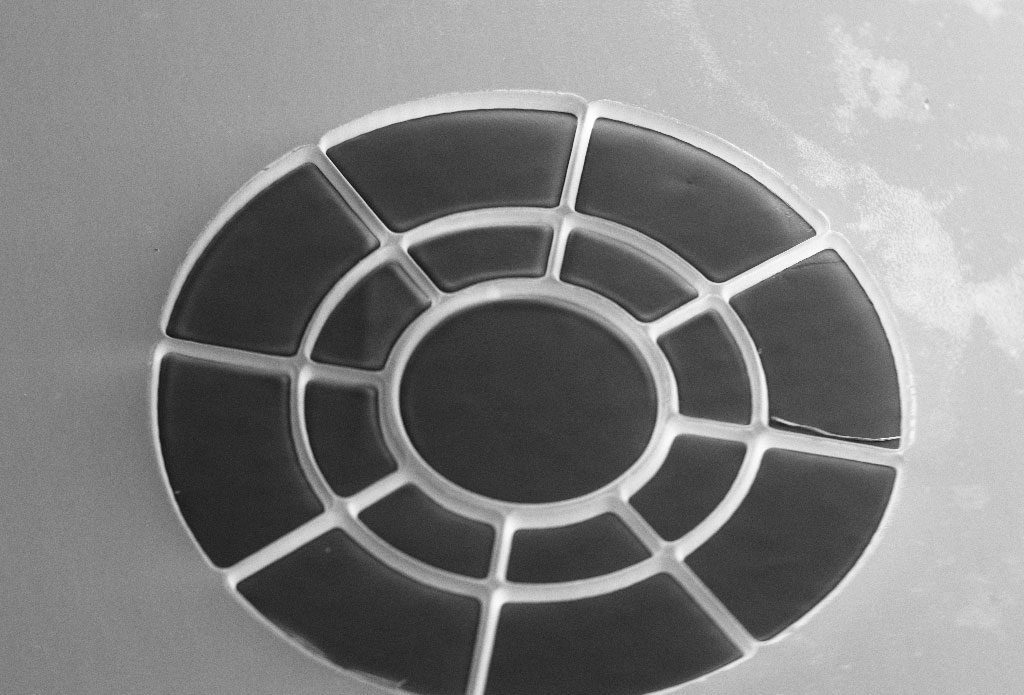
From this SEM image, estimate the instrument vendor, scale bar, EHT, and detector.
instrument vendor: Zeiss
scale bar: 100000 nm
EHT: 10 kV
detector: SE1